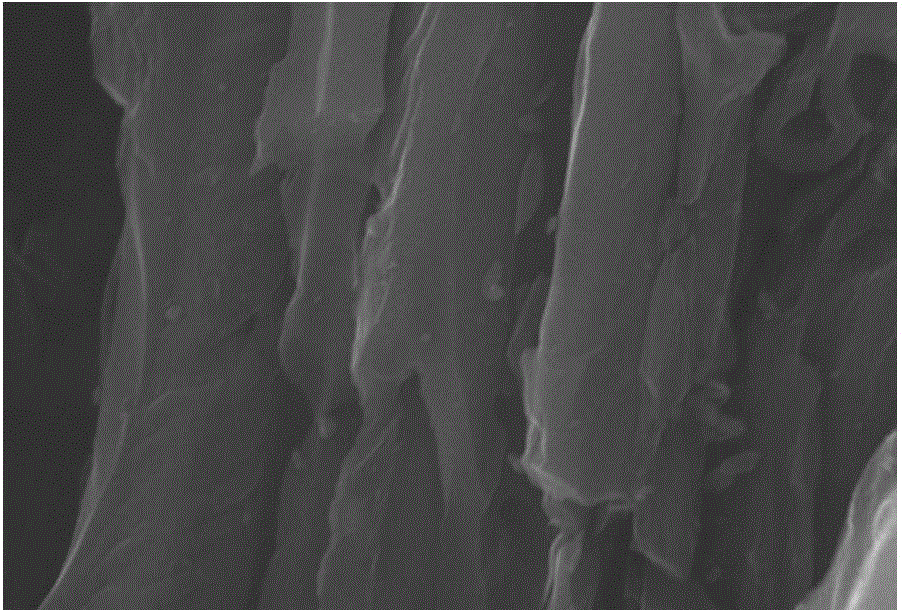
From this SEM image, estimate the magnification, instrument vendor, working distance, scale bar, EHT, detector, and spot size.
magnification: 6 K X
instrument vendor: JEOL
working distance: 10 mm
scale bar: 2000 nm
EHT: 30 kV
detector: SEI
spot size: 30